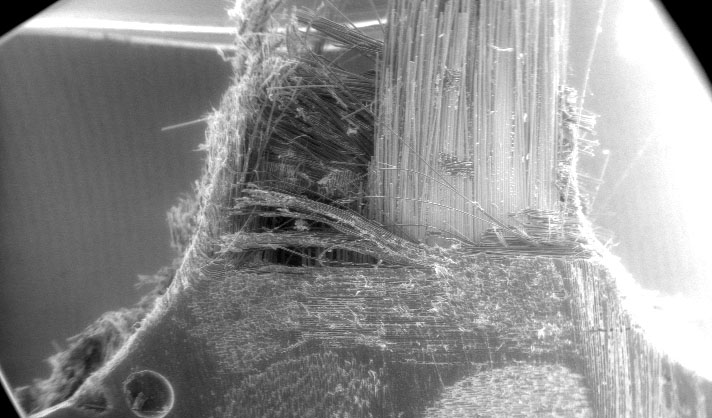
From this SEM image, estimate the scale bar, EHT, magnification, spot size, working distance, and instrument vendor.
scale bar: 1000000 nm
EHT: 12 kV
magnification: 0.022 K X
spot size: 5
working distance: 8.8 mm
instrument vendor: FEI